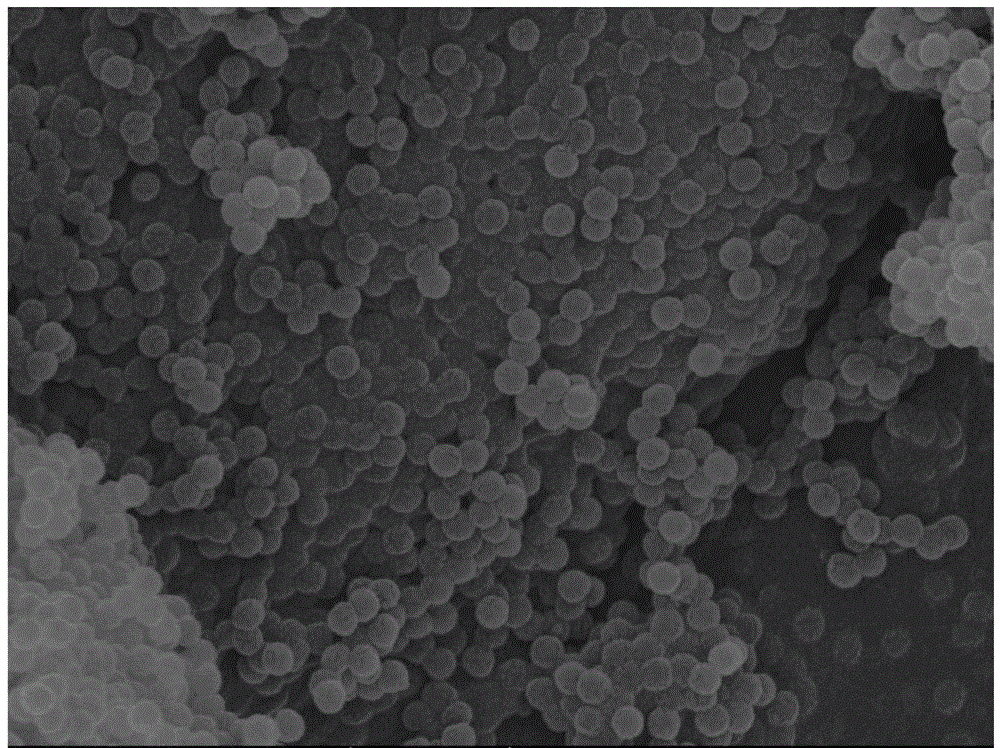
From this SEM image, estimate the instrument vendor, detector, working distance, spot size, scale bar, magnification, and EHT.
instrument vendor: FEI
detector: TLD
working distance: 4.6 mm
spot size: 3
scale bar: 2000 nm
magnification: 10 K X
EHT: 20 kV